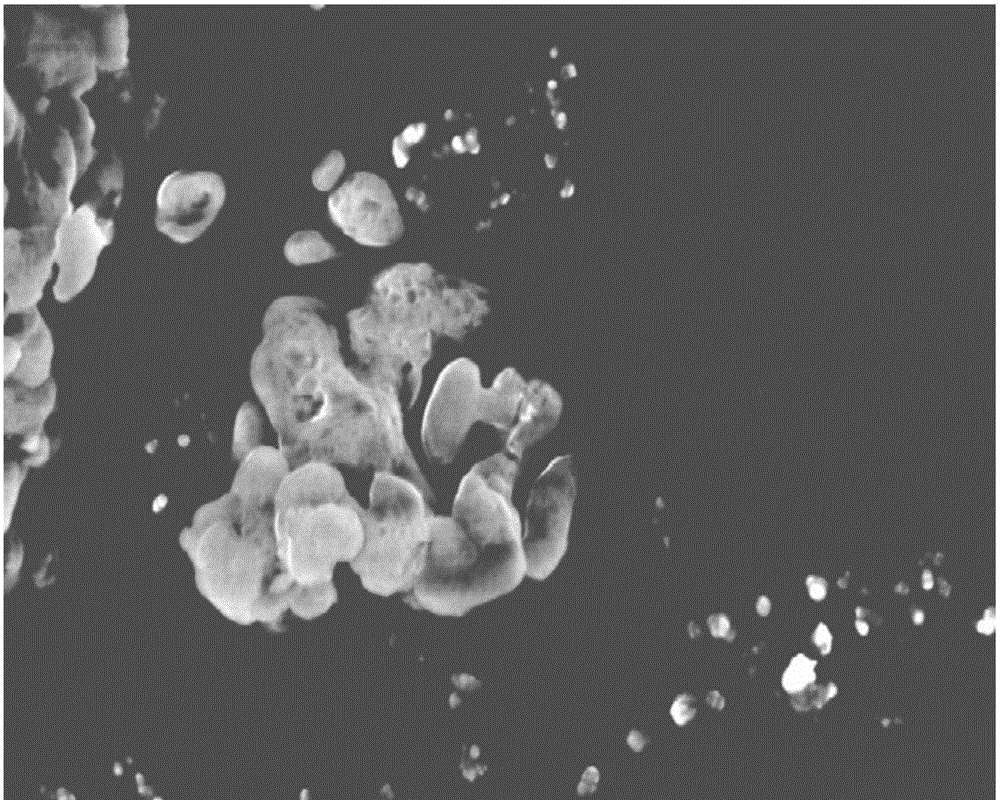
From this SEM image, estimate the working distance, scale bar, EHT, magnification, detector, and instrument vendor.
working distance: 4.7 mm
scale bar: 200 nm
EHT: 2 kV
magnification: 30 K X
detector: InlensDuo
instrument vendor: Zeiss